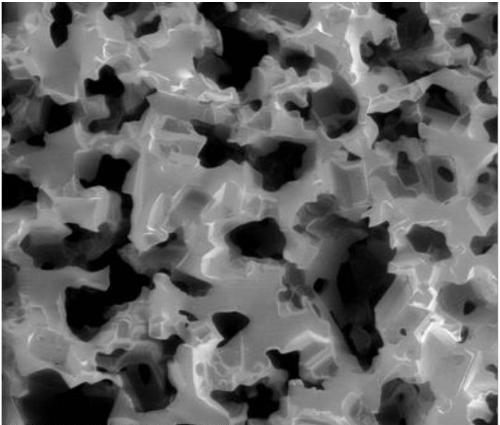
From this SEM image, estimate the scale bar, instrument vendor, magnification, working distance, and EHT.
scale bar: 1000000 nm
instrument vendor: FEI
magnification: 0.05 K X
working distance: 11.3 mm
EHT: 5 kV